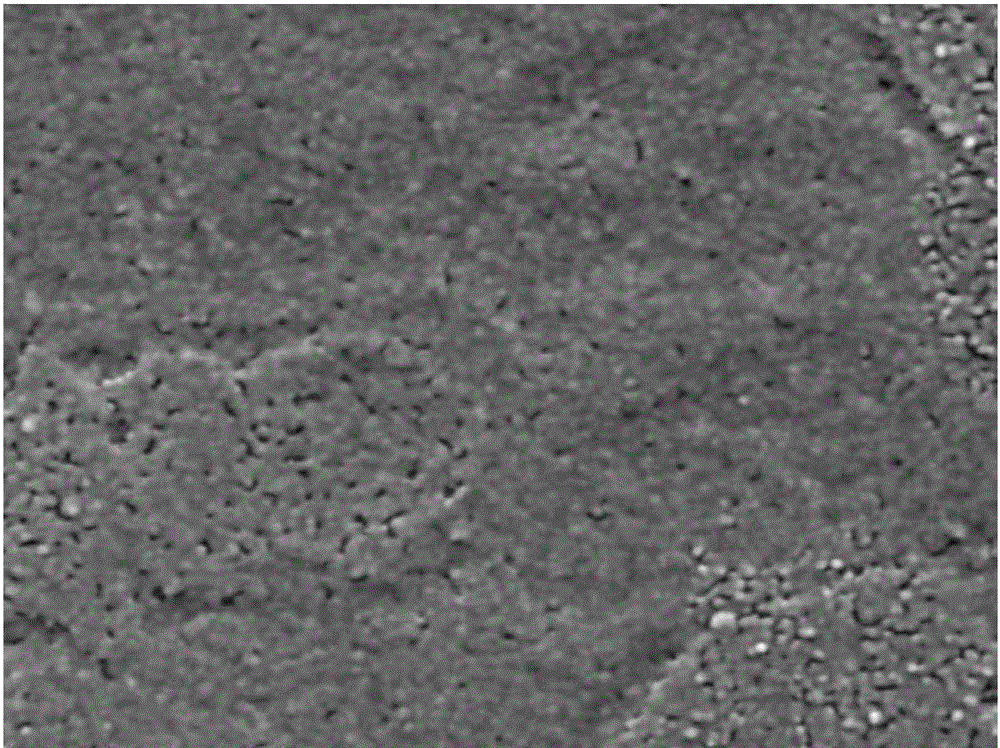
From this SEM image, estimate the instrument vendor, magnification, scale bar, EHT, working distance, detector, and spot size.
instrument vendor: FEI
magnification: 40 K X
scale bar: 500 nm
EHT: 20 kV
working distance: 17.6 mm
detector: SE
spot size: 5